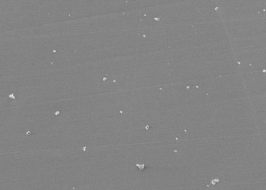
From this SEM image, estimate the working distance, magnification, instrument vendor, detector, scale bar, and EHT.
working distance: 11.5 mm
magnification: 0.5 K X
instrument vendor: JEOL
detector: SED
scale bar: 50000 nm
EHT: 10 kV